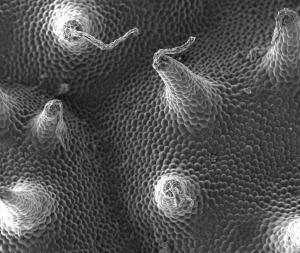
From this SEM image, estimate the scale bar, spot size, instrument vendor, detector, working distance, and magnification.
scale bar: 500000 nm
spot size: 3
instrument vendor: FEI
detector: ETD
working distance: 4.6 mm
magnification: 0.125 K X